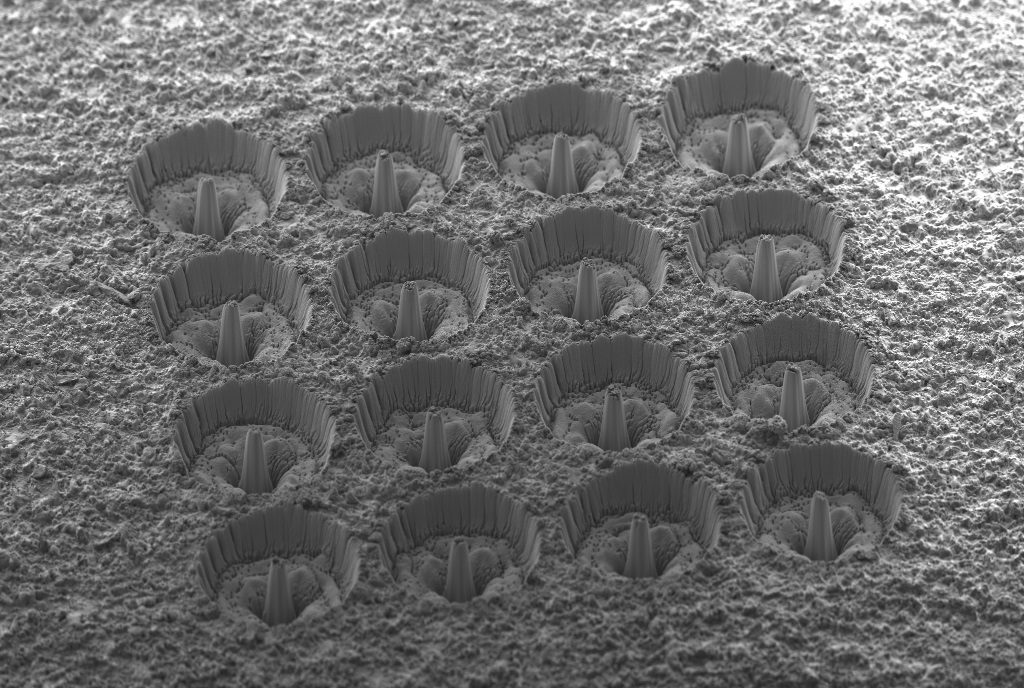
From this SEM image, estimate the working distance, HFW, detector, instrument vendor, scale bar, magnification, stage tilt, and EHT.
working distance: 5.7 mm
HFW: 1968 µm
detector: SESI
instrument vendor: Zeiss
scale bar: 200000 nm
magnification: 0.058 K X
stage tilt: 54°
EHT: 5 kV